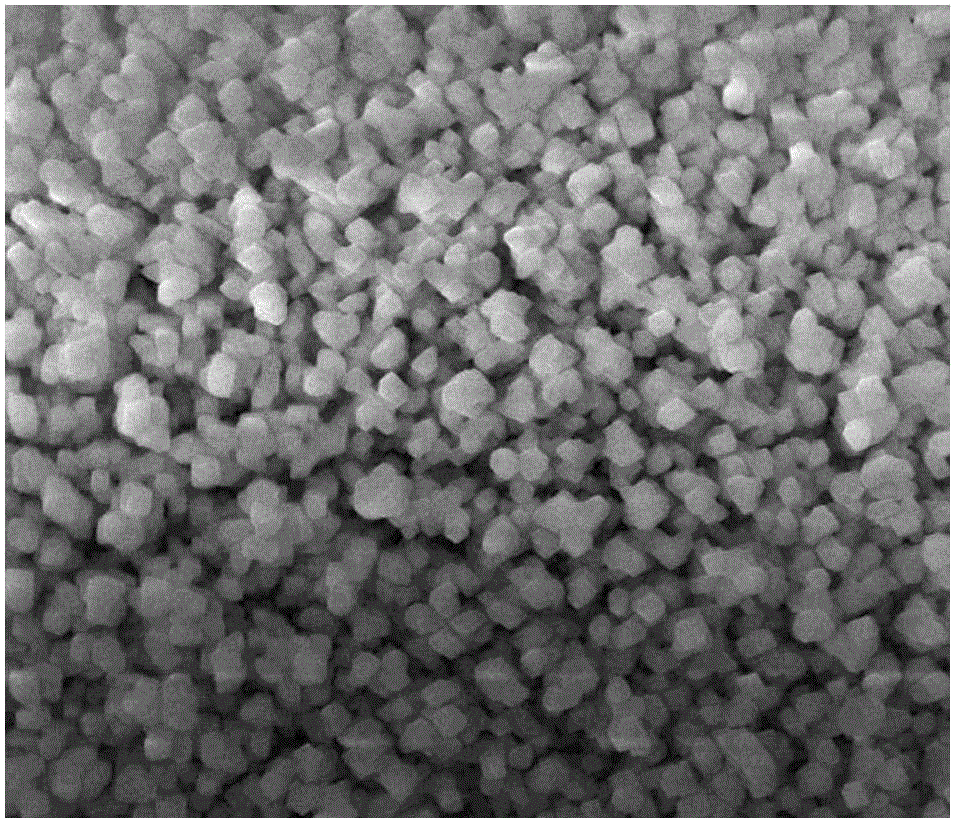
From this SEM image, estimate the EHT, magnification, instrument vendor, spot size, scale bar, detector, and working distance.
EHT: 15 kV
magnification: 30 K X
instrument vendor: FEI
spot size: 2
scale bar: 3000 nm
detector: ETD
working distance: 10.9 mm